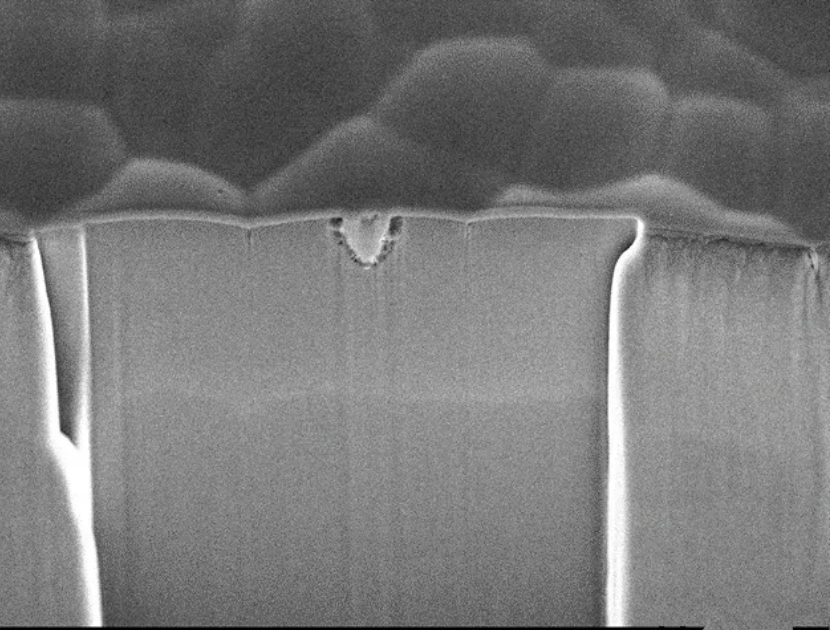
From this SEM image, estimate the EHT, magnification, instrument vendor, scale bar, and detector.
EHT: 10 kV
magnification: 7 K X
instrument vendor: JEOL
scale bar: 1000 nm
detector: SEI